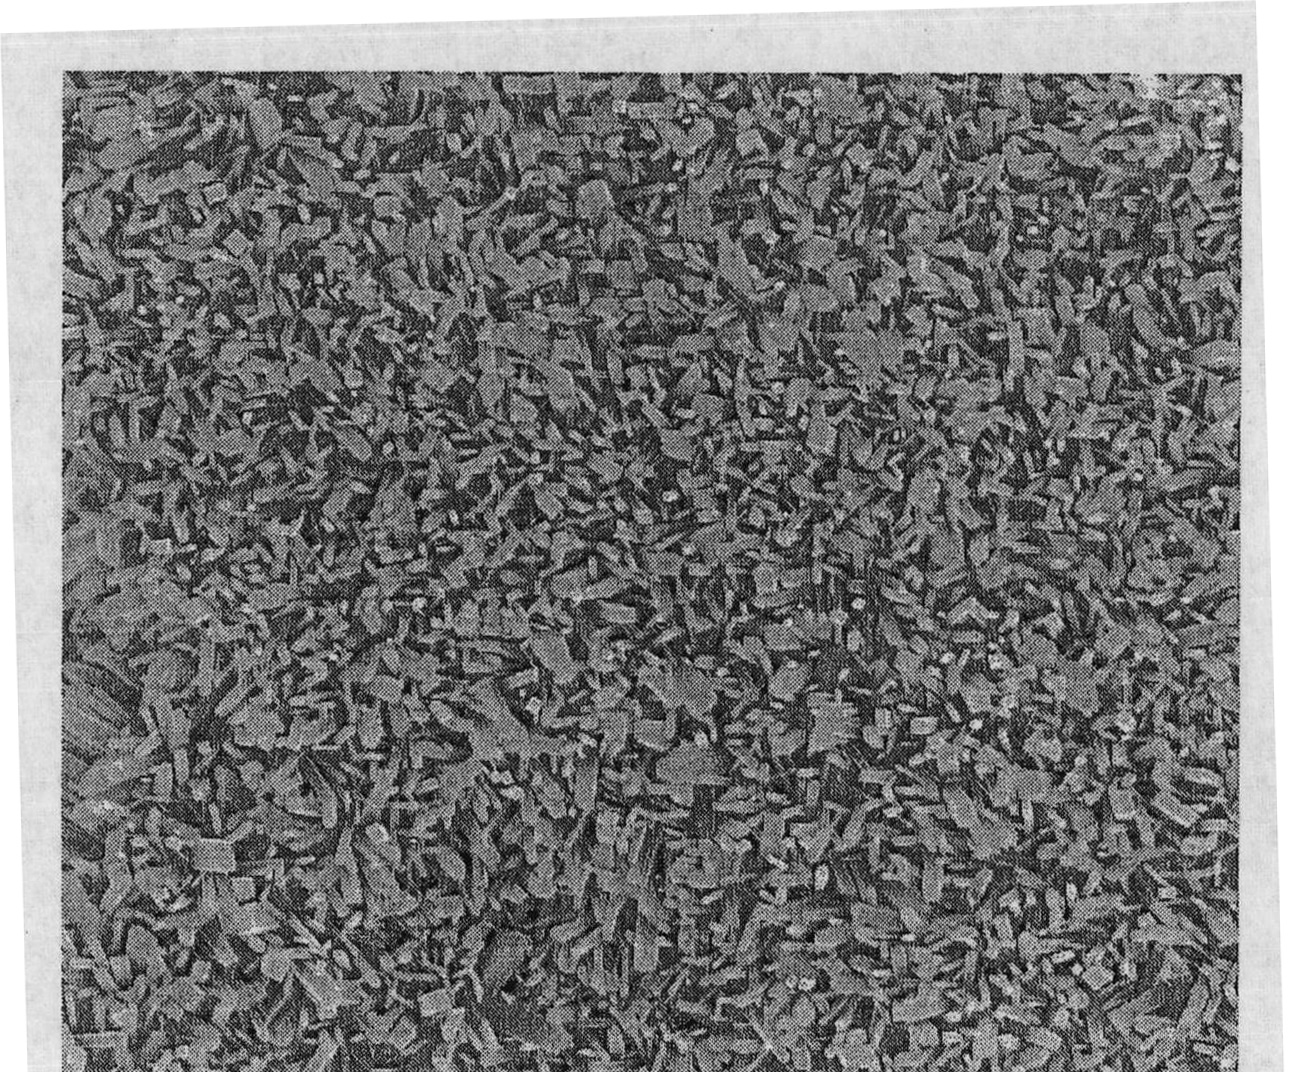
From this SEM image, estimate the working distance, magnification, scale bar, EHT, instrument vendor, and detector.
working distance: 6.3 mm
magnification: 50 K X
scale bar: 1000 nm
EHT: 10 kV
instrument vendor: FEI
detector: TLD